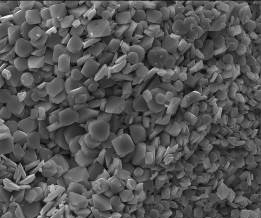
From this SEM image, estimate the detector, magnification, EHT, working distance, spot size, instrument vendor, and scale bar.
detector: ETD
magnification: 1.75 K X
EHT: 30 kV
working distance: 10.9 mm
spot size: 3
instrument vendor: FEI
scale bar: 20000 nm